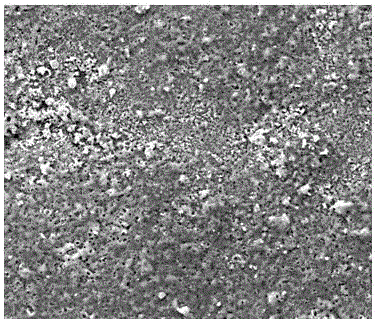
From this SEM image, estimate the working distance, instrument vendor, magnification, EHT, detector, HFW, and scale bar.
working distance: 9.1 mm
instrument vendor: FEI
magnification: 1.5 K X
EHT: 20 kV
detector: ETD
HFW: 170 µm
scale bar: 50000 nm